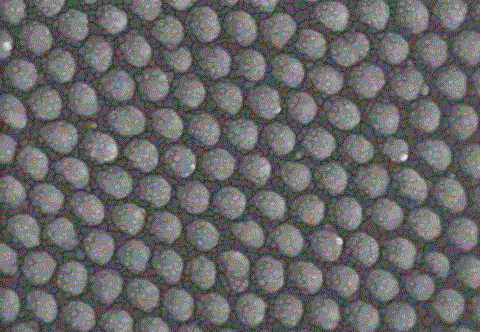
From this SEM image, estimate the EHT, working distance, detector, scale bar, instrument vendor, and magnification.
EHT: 10 kV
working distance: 10.1 mm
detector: SE(M)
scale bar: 500 nm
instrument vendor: Hitachi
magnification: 100 K X